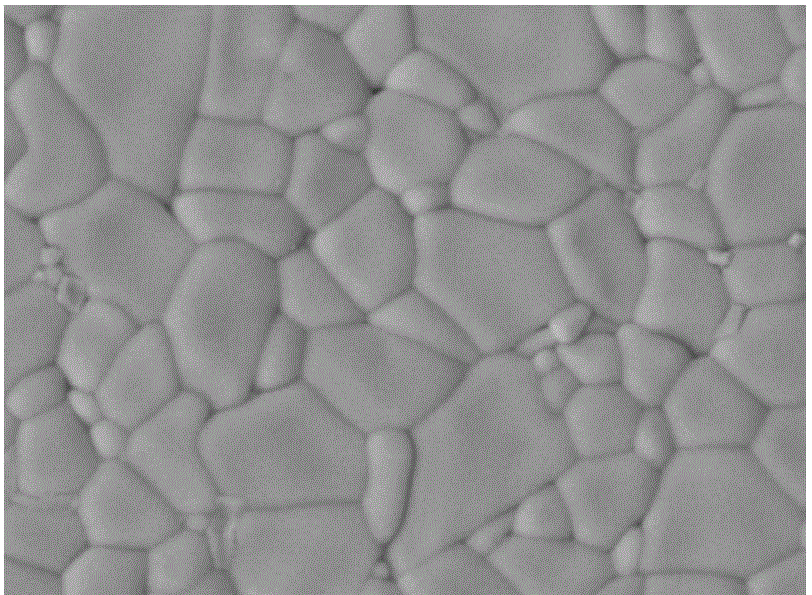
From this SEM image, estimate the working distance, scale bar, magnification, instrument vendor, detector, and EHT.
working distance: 11 mm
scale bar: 10000 nm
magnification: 2 K X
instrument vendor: JEOL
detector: COMP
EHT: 20 kV